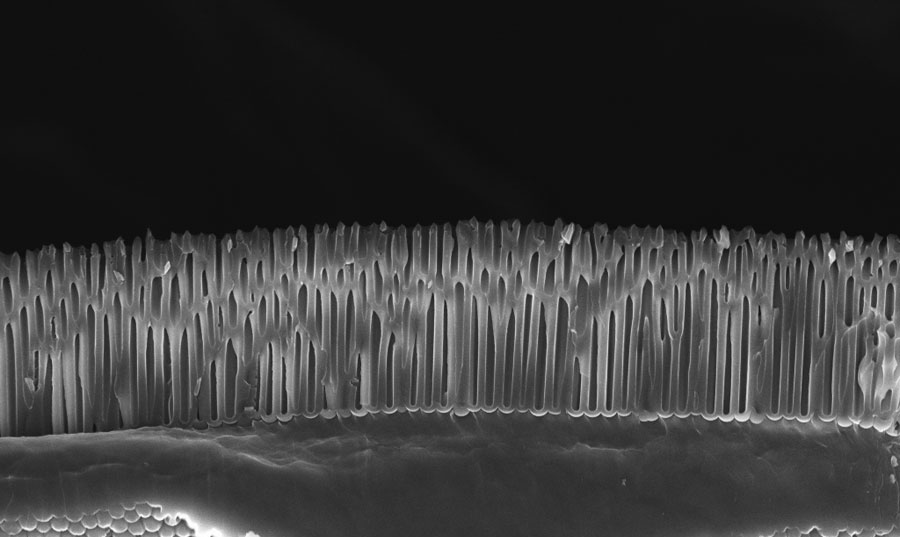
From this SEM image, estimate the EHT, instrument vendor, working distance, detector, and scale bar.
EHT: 10 kV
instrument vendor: Zeiss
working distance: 4 mm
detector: InLens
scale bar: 2000 nm